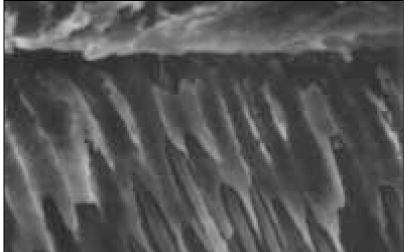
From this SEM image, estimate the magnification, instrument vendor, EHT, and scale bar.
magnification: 2 K X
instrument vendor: Hitachi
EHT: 20 kV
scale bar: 20000 nm